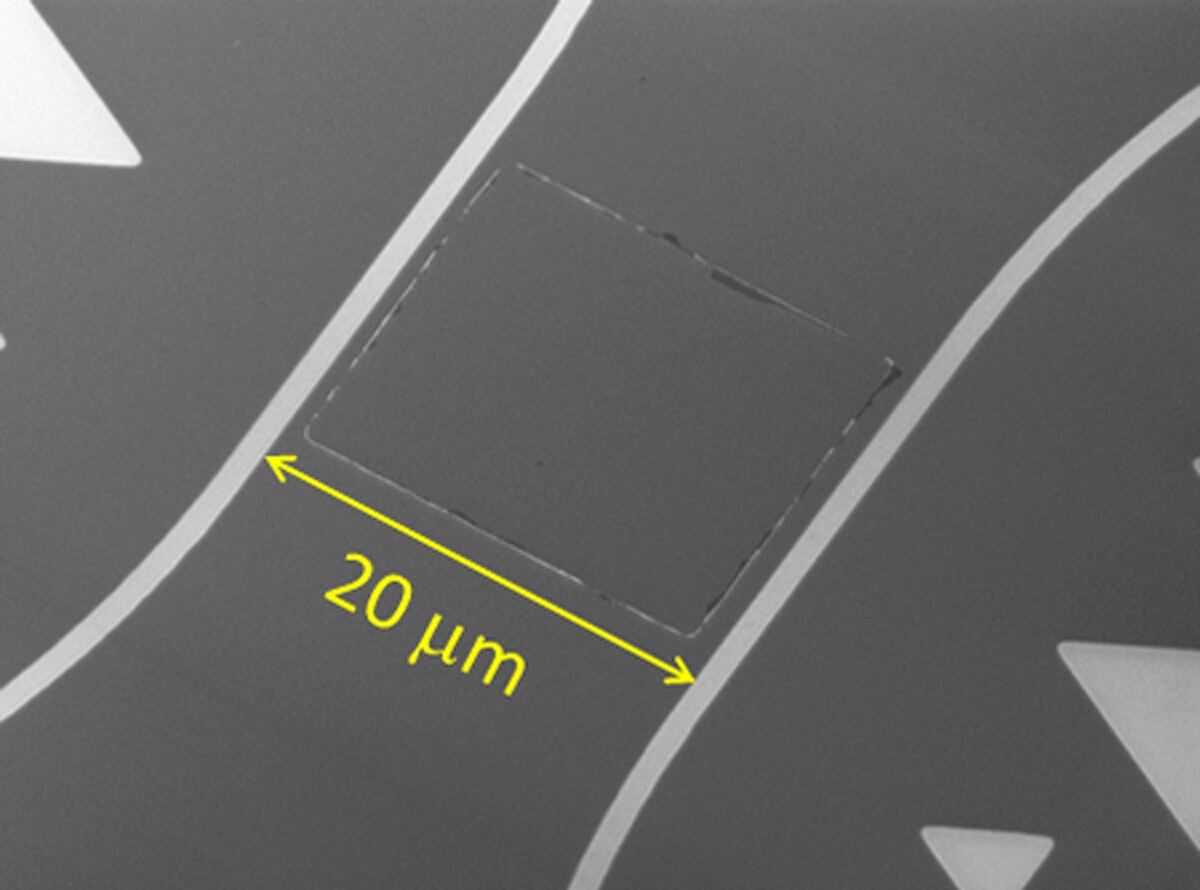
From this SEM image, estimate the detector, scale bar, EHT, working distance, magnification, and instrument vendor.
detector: SEI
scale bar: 10000 nm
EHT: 5 kV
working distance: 8.1 mm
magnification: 2.5 K X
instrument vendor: JEOL